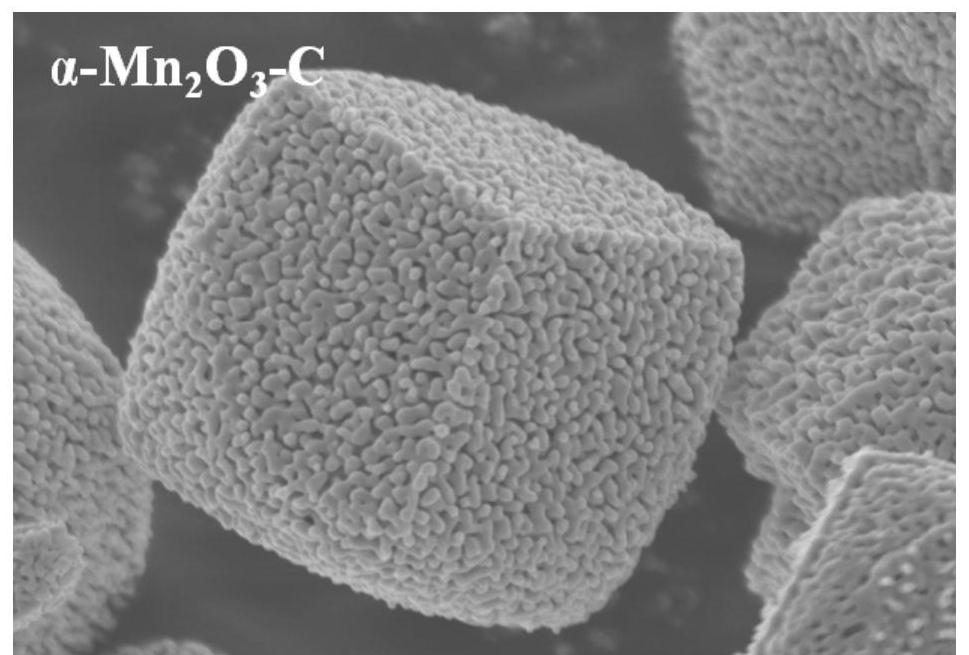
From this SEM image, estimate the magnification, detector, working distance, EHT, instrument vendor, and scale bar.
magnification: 25 K X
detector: SE(UL)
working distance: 7.3 mm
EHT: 3 kV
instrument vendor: Hitachi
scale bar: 2000 nm